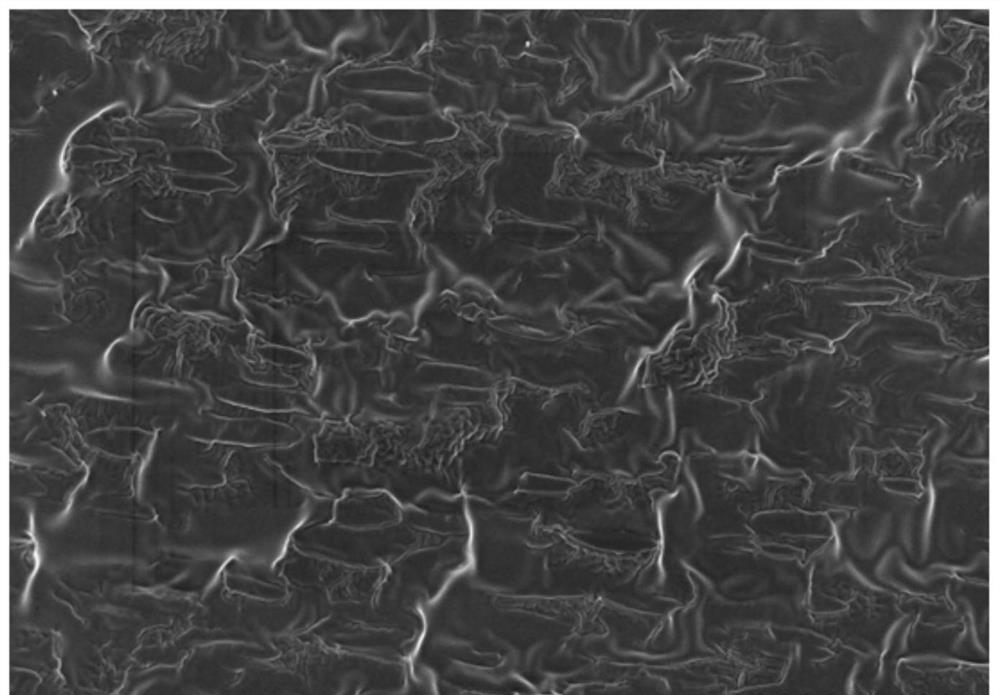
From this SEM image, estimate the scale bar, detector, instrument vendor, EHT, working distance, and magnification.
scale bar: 10000 nm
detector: SE(M)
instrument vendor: Hitachi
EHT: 3 kV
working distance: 7.6 mm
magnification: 3 K X